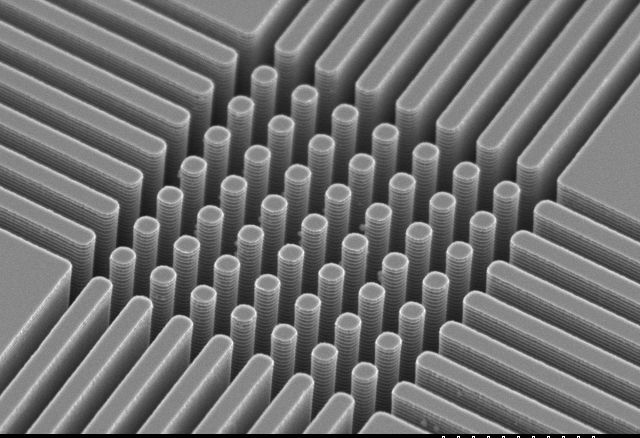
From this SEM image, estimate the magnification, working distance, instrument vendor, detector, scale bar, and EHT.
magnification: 3 K X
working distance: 11 mm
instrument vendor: Hitachi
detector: SE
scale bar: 10000 nm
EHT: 5 kV